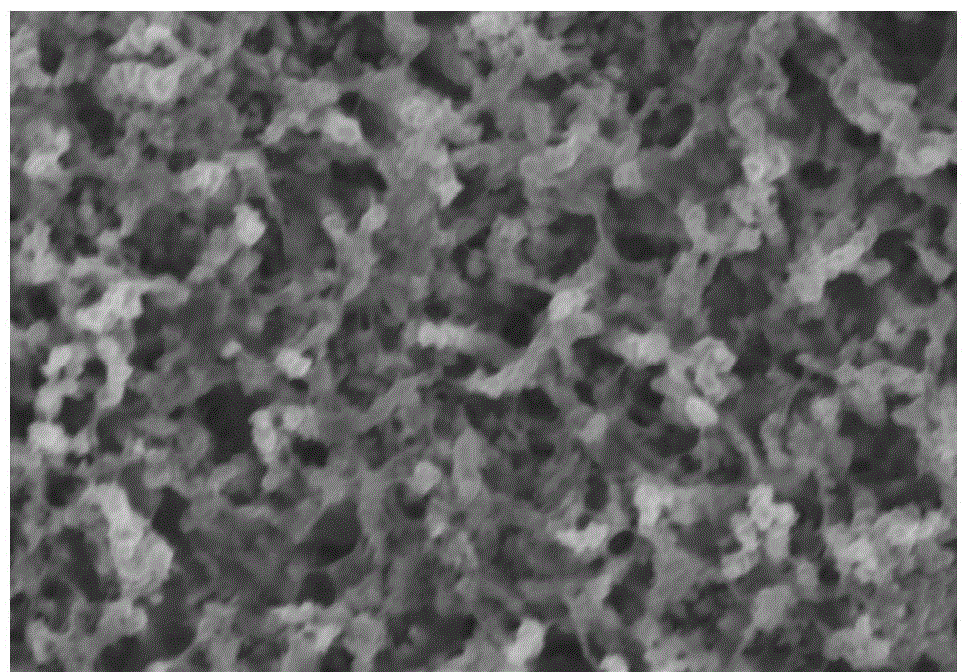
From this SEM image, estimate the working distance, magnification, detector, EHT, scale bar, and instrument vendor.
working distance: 5.8 mm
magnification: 50 K X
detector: SE(U)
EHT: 3 kV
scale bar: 1000 nm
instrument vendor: Hitachi